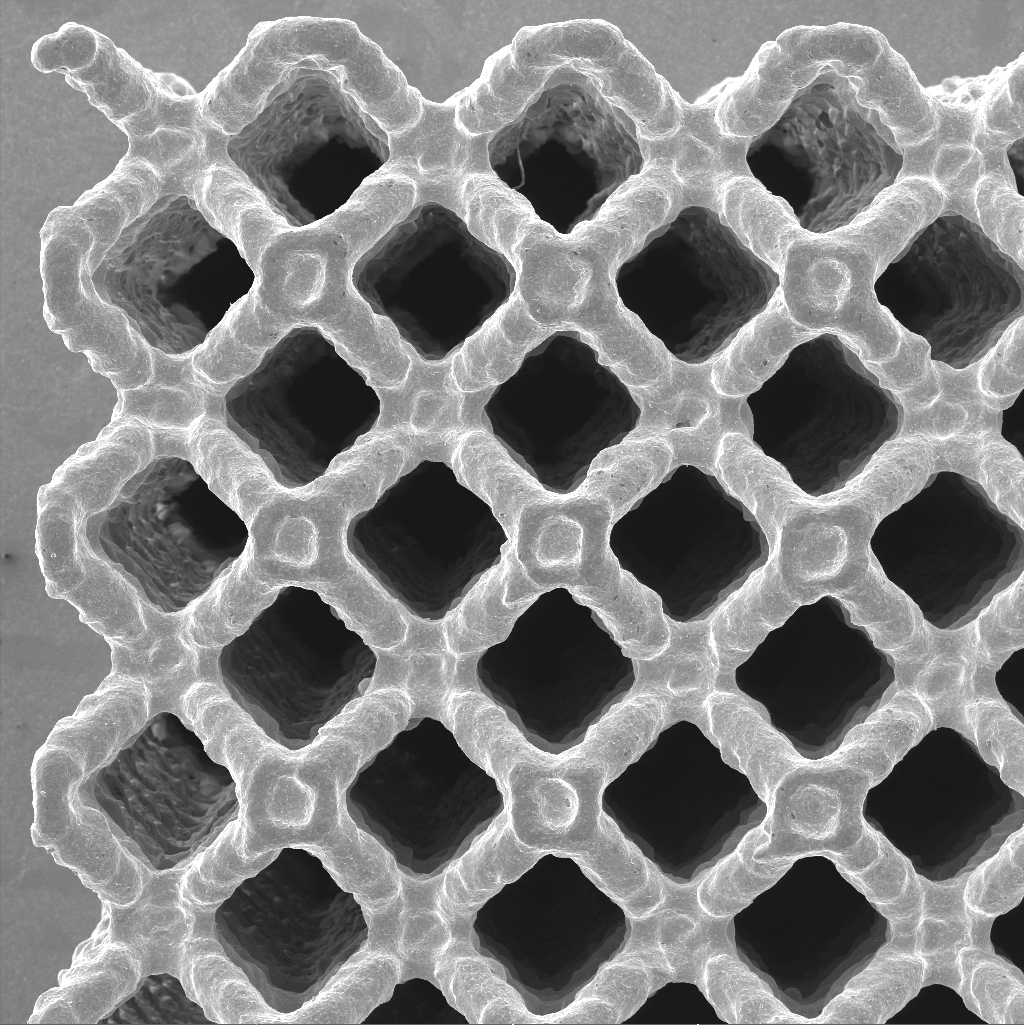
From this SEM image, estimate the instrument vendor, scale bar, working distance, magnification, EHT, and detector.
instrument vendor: Tescan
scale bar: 1000000 nm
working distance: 41.26 mm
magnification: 0.05 K X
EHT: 20 kV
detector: SE